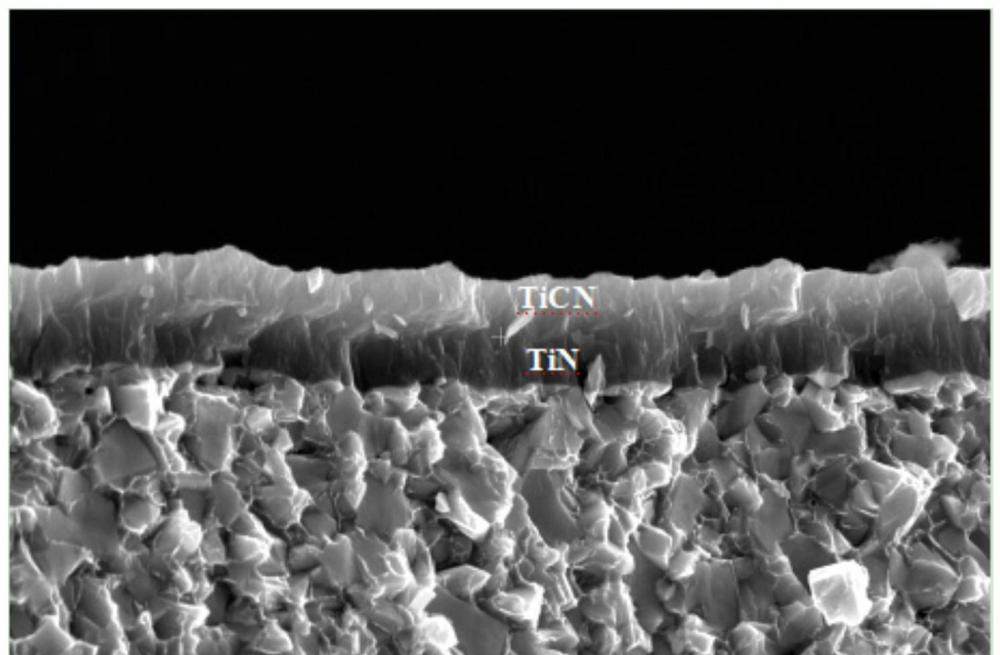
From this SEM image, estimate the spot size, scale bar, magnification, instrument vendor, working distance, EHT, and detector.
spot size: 8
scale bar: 1000 nm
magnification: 50 K X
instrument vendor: Thermo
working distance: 10.3596 mm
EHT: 15 kV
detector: ETD